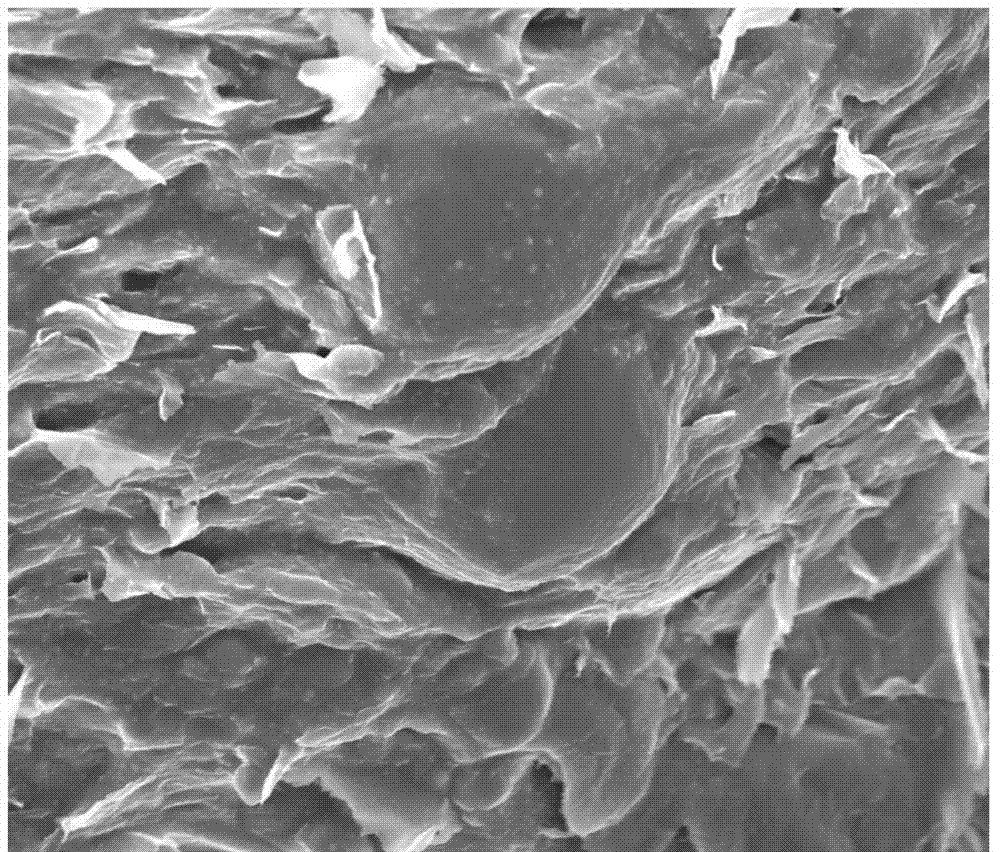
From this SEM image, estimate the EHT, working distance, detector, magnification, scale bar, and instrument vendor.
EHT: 15 kV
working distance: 6.2 mm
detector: TLD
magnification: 5 K X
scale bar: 10000 nm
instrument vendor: FEI